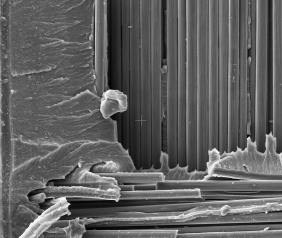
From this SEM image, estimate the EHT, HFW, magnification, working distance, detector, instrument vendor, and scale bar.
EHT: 10 kV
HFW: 149 µm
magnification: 1 K X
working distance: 5 mm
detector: ETD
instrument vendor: FEI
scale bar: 50000 nm